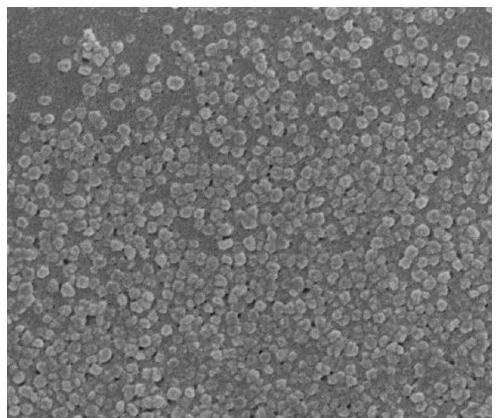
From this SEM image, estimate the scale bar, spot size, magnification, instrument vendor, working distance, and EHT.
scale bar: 1000 nm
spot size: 3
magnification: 80 K X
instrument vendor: FEI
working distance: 5.1 mm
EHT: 3 kV